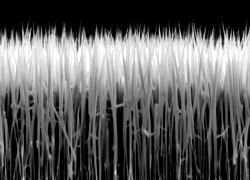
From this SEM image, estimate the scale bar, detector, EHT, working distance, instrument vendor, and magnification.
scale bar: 1000 nm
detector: SEI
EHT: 5 kV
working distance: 6 mm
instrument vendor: JEOL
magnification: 16 K X